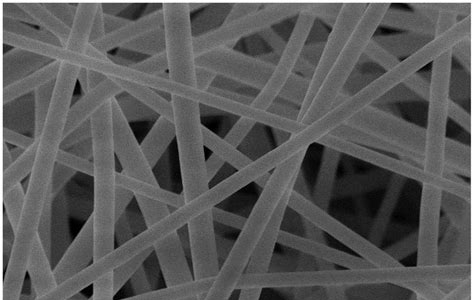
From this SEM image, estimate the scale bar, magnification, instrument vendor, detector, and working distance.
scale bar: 5000 nm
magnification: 10 K X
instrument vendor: Hitachi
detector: SE(M)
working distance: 6.8 mm